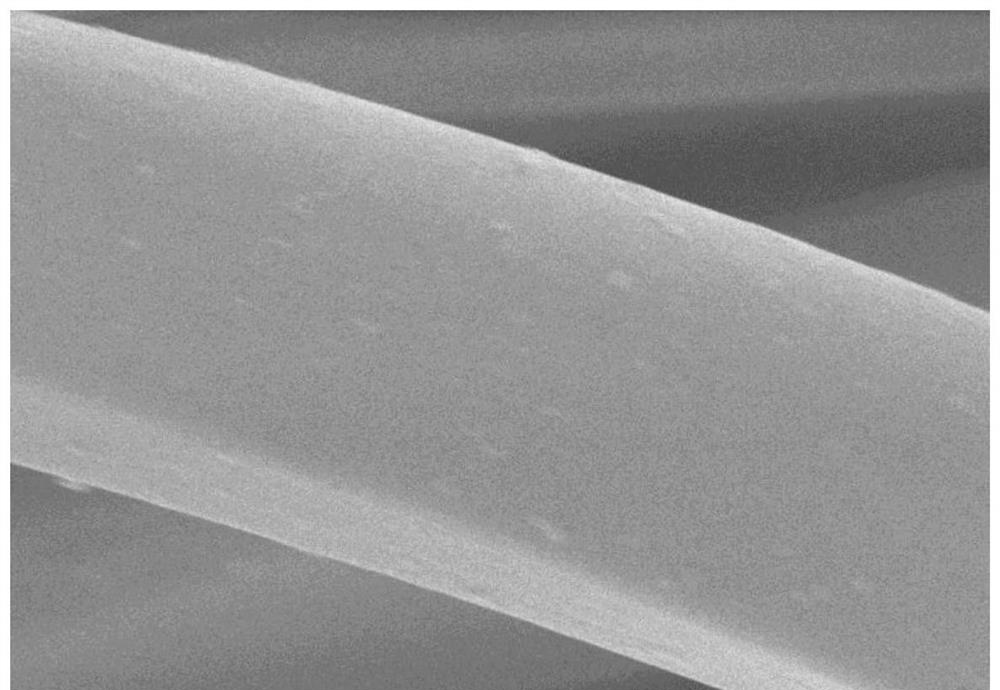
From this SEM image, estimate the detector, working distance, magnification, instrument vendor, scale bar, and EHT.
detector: SE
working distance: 8.5 mm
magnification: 5 K X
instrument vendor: Hitachi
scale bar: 10000 nm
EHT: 5 kV